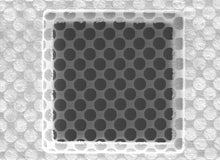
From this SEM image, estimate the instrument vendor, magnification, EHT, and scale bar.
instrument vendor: other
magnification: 1.65 K X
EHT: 5 kV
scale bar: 20000 nm